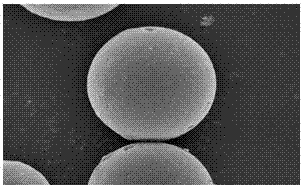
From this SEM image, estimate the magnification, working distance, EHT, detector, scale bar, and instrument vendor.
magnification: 10 K X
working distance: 5 mm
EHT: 3 kV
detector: SE(UL)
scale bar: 5000 nm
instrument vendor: Hitachi